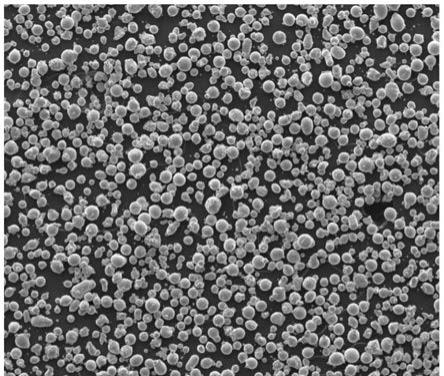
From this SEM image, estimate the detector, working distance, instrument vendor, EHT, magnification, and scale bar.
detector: ETD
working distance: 10 mm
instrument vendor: FEI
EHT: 10 kV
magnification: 0.2 K X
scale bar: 500000 nm